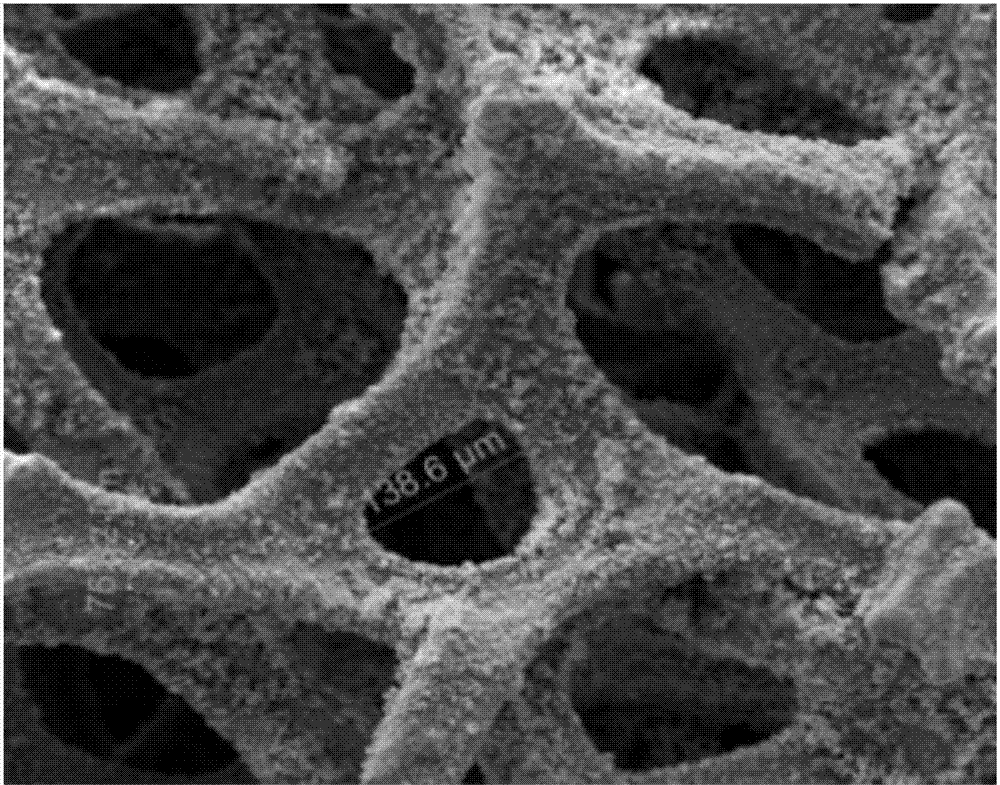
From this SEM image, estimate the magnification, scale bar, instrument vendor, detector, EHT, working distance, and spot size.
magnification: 0.4 K X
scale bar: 200000 nm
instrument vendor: FEI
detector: ETD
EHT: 15 kV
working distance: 10.7 mm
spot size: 5